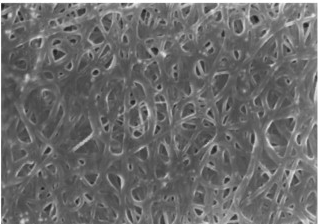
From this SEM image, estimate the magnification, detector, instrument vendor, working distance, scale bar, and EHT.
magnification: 50 K X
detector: SE(M)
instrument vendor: Hitachi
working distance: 11.9 mm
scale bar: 1000 nm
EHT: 5 kV